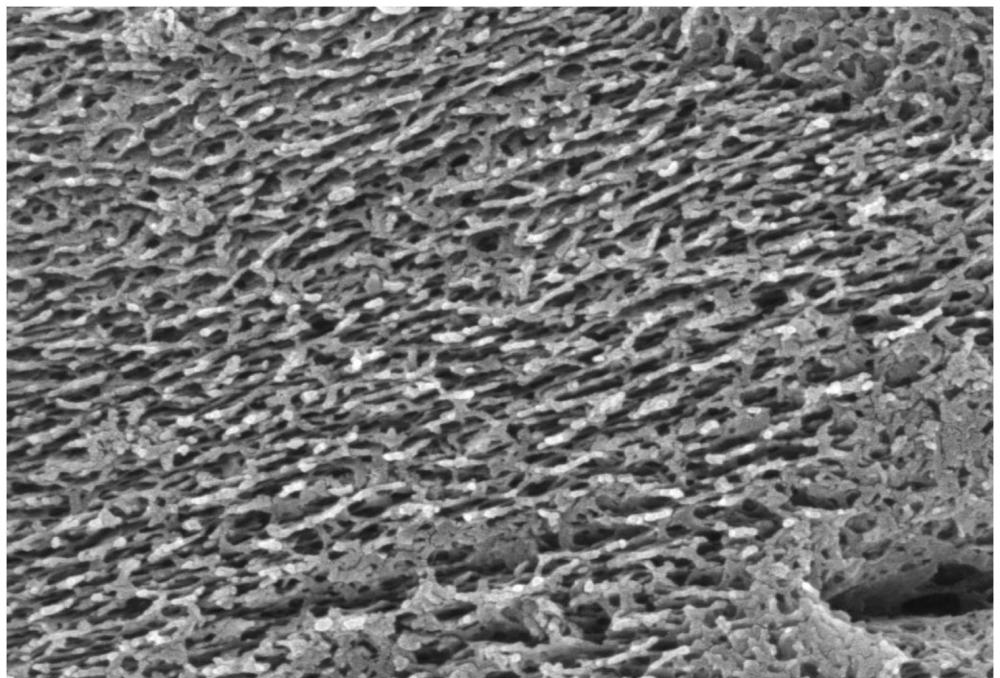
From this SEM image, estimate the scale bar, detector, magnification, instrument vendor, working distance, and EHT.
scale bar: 200 nm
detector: InLens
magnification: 50 K X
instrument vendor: Zeiss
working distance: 4.2 mm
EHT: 3 kV